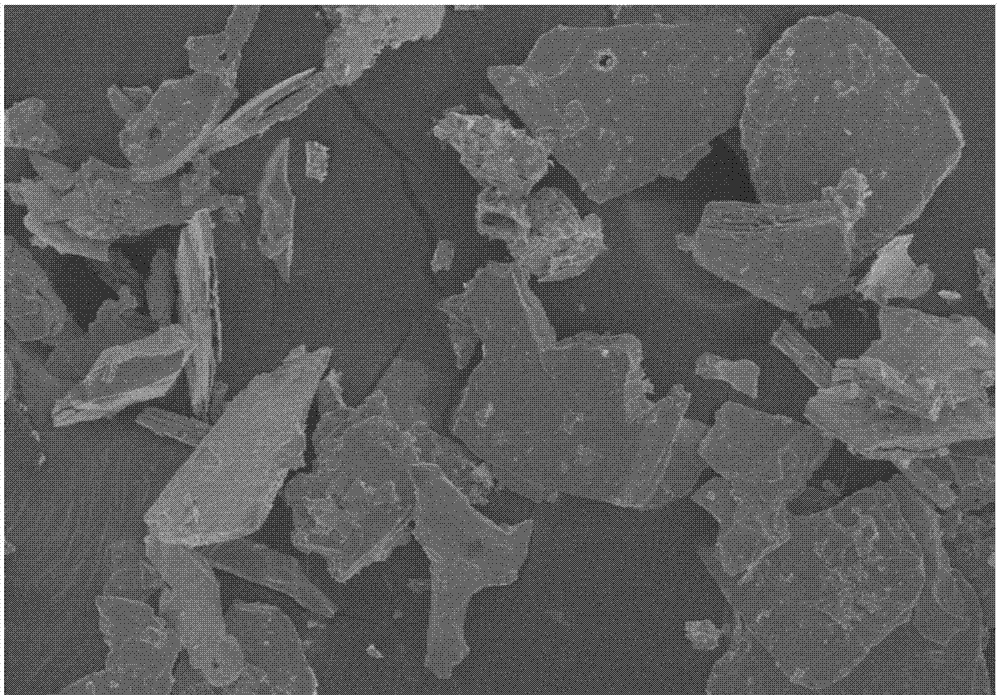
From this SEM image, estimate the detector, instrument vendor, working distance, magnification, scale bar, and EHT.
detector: SE(M)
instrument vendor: Hitachi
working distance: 8.6 mm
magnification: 0.4 K X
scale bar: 100000 nm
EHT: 5 kV